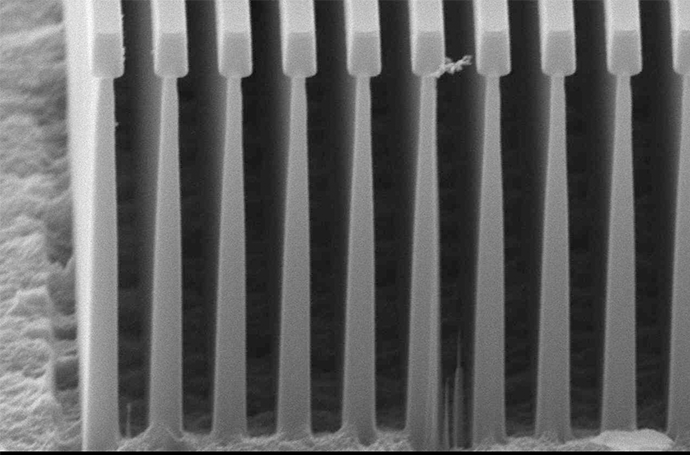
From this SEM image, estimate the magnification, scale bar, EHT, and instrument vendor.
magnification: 8 K X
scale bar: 2000 nm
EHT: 15 kV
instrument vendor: JEOL